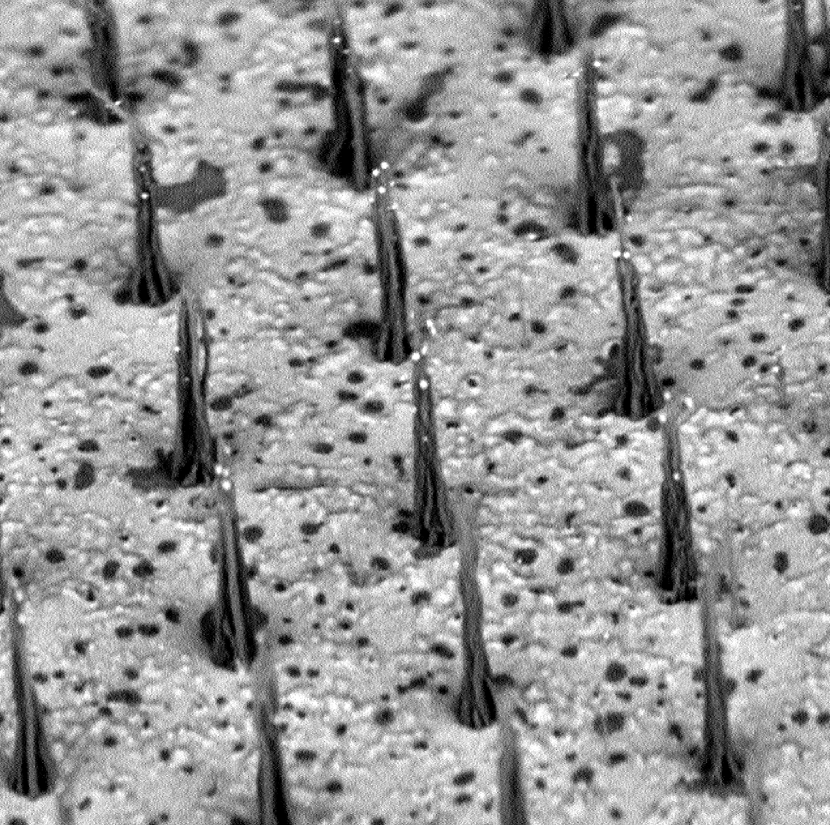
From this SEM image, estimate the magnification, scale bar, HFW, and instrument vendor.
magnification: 8.1 K X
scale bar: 10000 nm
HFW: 24.7 µm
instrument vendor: FEI